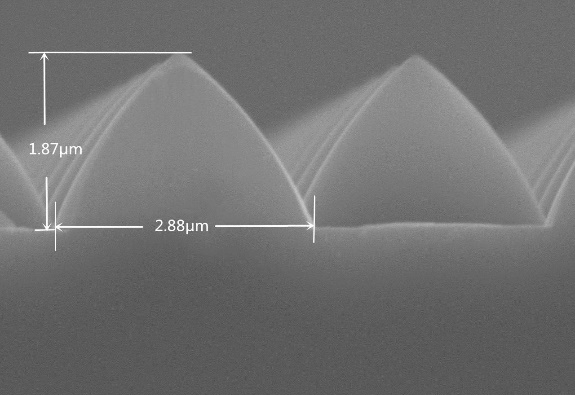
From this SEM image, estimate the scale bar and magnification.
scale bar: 2000 nm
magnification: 20 K X